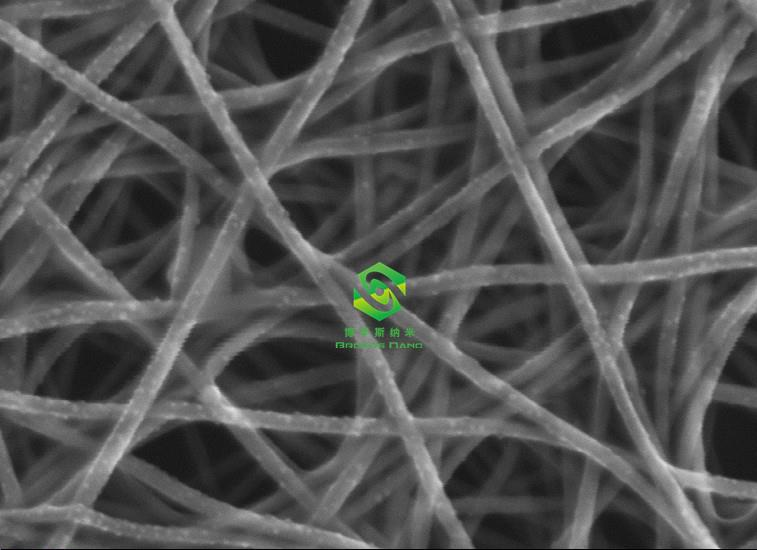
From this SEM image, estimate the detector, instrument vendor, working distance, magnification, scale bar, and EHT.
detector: InLens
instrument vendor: Zeiss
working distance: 2.9 mm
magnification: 200 K X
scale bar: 100 nm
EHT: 20 kV